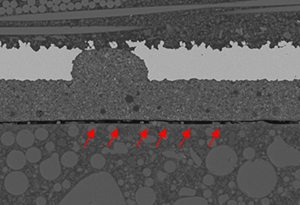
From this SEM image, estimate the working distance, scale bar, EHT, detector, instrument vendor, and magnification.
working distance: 10.1 mm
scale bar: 50000 nm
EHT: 15 kV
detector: BSE3D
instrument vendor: Hitachi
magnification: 0.75 K X